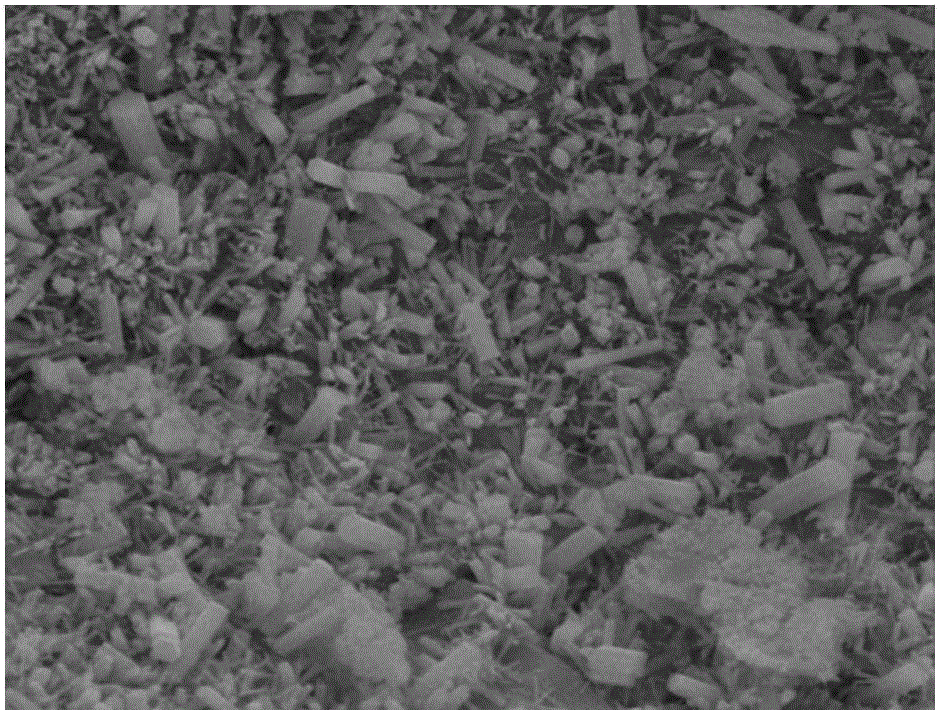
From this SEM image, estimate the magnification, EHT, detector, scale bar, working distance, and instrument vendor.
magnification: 5 K X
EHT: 20 kV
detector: ETD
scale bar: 20000 nm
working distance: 12.5 mm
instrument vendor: FEI